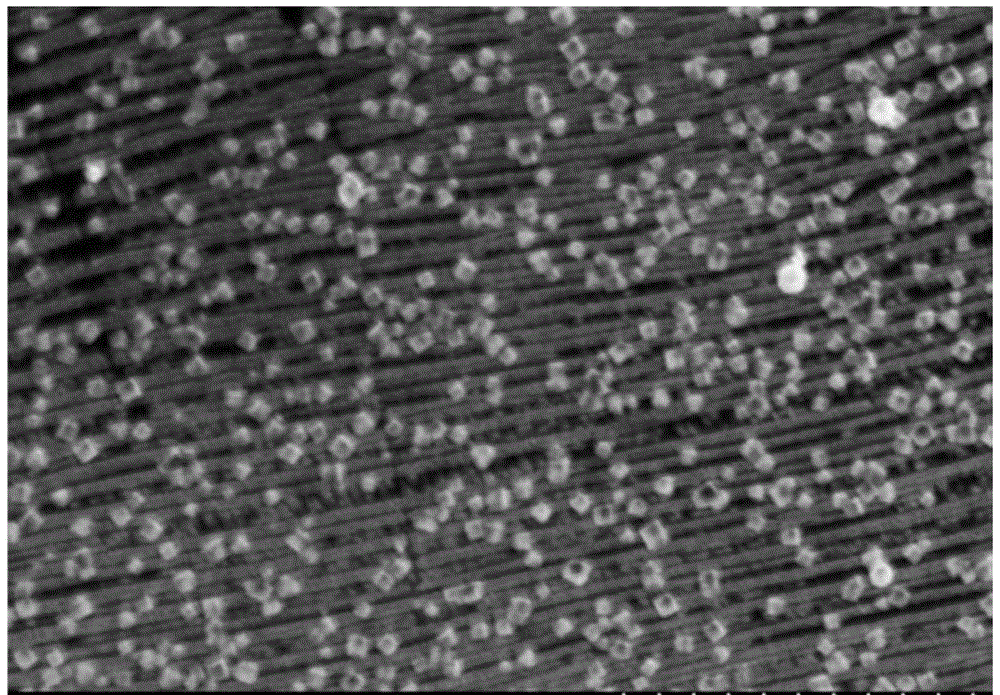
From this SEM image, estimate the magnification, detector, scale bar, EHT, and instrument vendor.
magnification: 15 K X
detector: SE(M)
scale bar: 3000 nm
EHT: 4 kV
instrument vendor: Hitachi